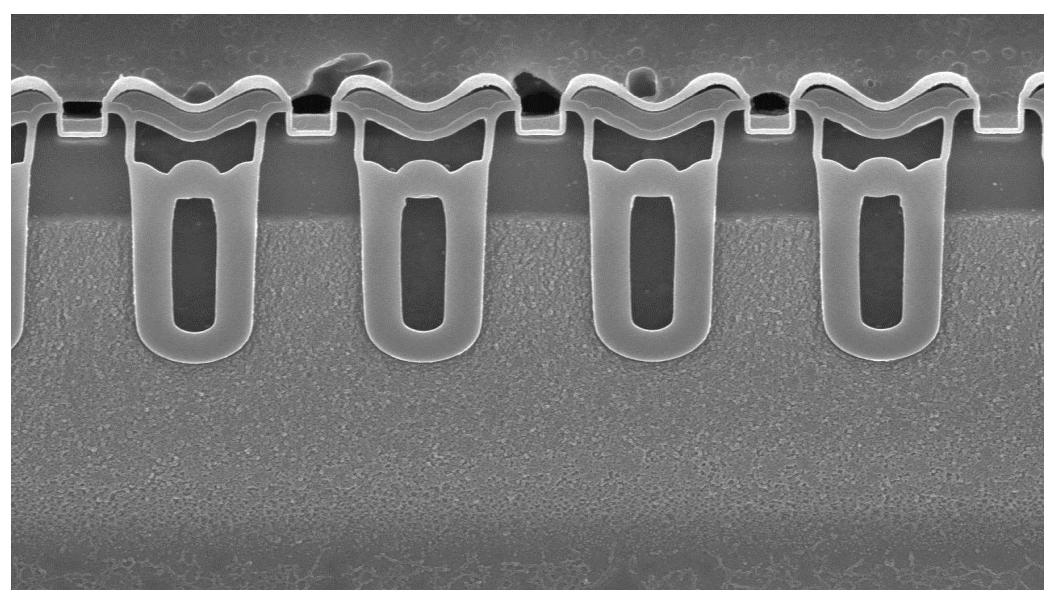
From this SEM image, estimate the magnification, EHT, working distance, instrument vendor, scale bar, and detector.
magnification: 8 K X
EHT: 15 kV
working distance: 8.1 mm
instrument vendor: Hitachi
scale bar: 5000 nm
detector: SE(U)